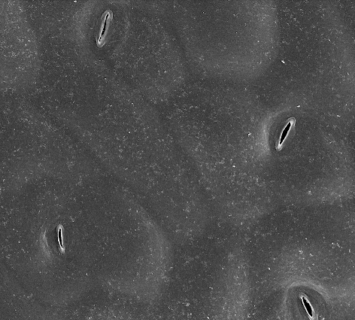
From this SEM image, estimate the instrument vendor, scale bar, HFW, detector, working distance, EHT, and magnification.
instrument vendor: FEI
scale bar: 40000 nm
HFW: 149 µm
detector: ETD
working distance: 10 mm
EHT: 5 kV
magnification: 1 K X